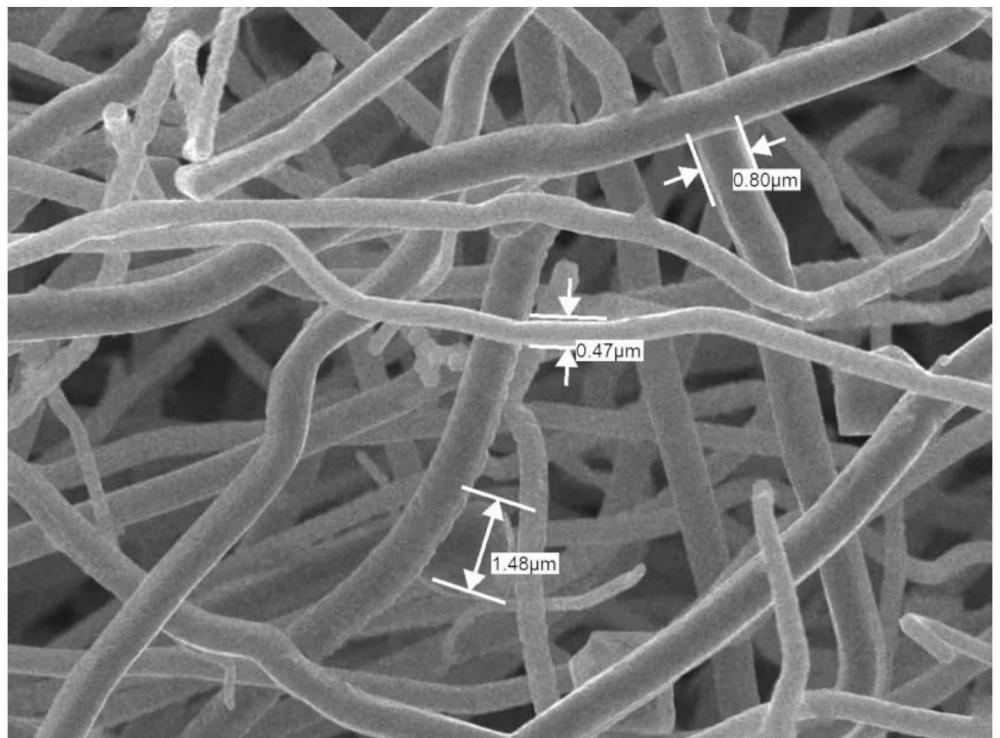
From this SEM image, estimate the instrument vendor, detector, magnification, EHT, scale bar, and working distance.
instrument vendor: JEOL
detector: SEI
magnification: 8 K X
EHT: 15 kV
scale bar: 1000 nm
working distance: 7.9 mm